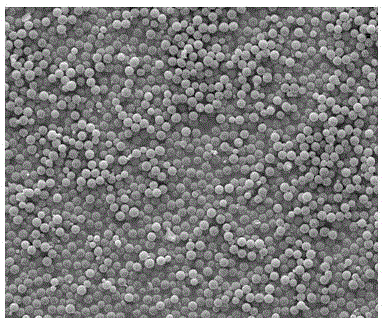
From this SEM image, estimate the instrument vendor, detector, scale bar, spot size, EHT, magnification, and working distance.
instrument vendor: FEI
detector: ETD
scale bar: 20000 nm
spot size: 3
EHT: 12.5 kV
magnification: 2 K X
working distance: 10.9 mm